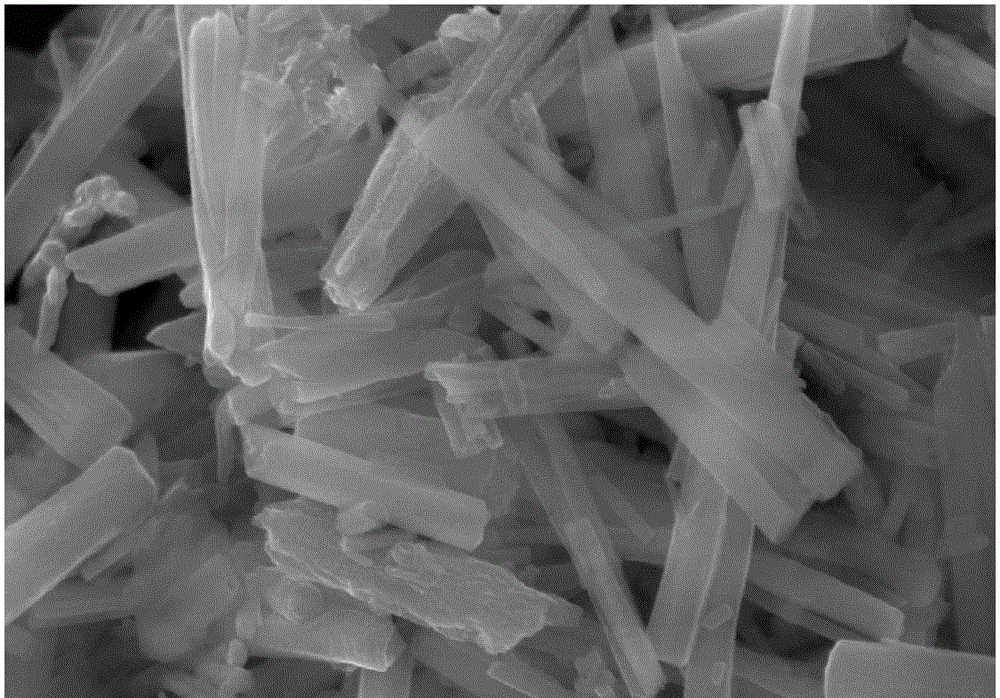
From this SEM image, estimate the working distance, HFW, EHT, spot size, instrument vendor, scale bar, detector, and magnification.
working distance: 9.3 mm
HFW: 5.18 µm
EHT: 20 kV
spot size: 3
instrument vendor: FEI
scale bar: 2000 nm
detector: ETD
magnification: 80 K X